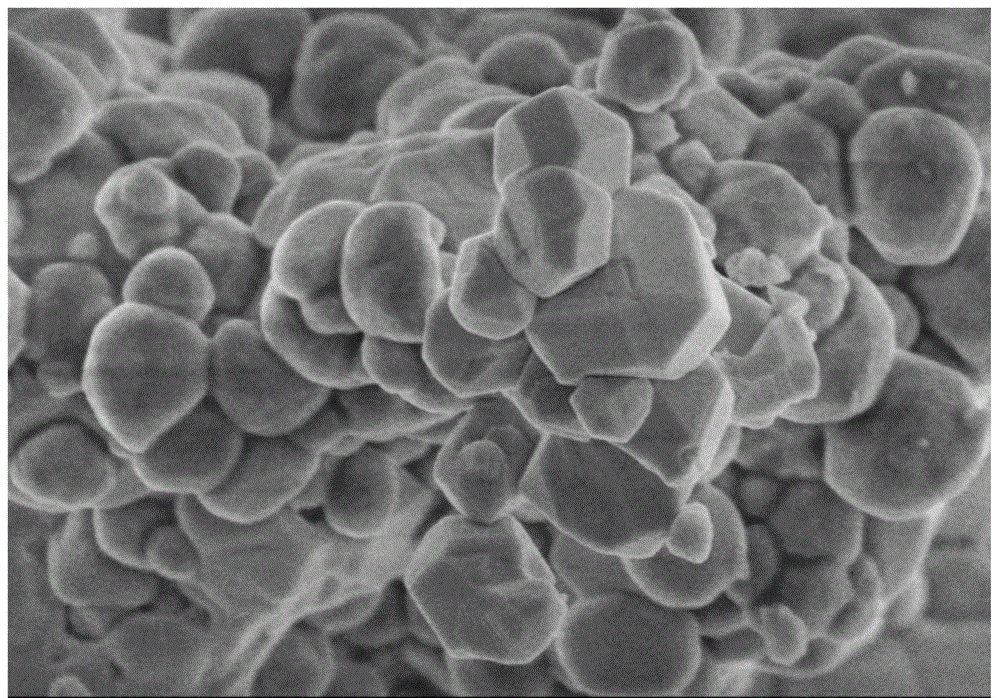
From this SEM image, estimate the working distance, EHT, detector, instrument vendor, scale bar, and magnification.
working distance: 8.8 mm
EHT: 3 kV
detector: SE(M)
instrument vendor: Hitachi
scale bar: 1000 nm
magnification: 30 K X